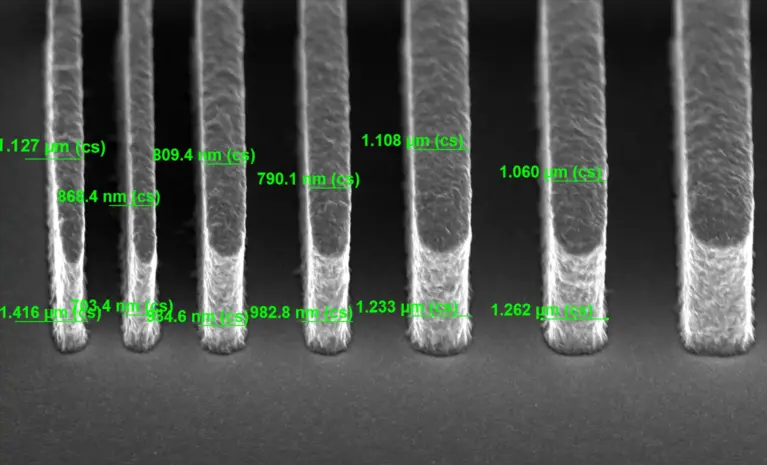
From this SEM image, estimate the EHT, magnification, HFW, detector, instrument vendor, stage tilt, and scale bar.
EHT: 5 kV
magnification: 14 K X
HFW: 14.8 µm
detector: TLD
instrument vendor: FEI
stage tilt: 52°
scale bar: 4000 nm